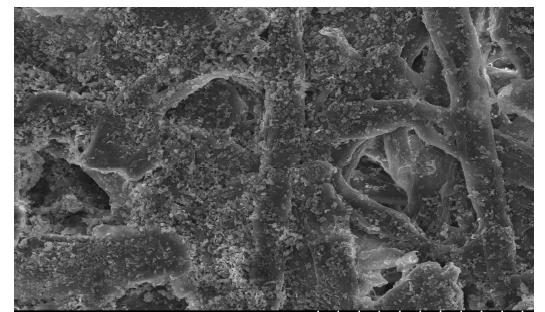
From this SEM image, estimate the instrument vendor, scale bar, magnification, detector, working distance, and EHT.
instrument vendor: Hitachi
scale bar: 50000 nm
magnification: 1 K X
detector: SE(U)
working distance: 12 mm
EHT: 15 kV